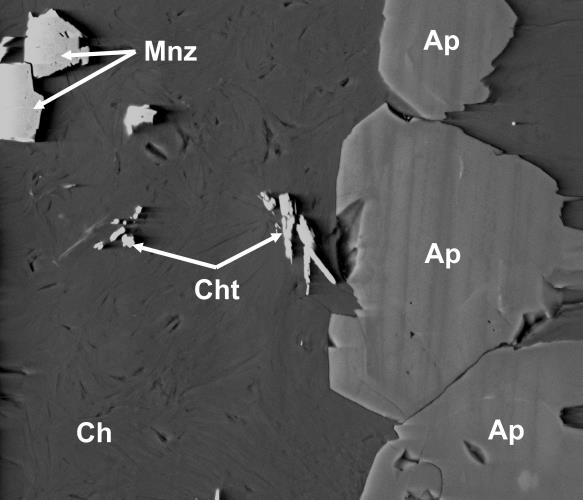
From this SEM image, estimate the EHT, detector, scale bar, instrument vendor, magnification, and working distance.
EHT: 15 kV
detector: SSD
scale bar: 50000 nm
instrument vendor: FEI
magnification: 3 K X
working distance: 9.9 mm